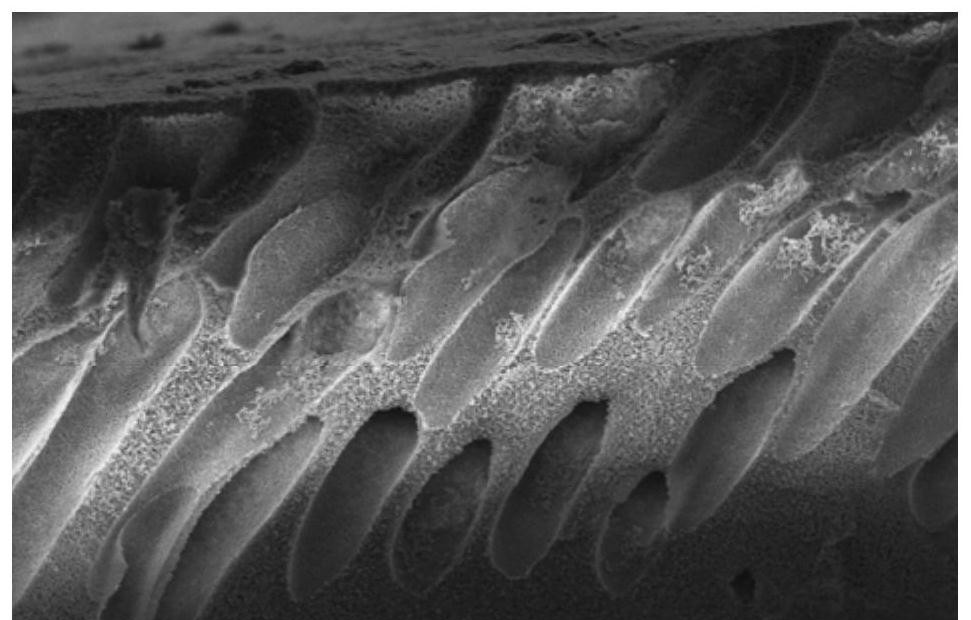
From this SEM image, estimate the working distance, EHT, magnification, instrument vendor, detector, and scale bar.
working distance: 8.6 mm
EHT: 5 kV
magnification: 0.9 K X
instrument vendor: Hitachi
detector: SE(M)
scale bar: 50000 nm